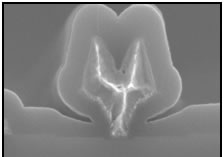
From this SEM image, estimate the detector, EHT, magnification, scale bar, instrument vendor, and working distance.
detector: SE(U)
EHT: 10 kV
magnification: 50 K X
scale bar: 1000 nm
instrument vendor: Hitachi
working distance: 11.4 mm